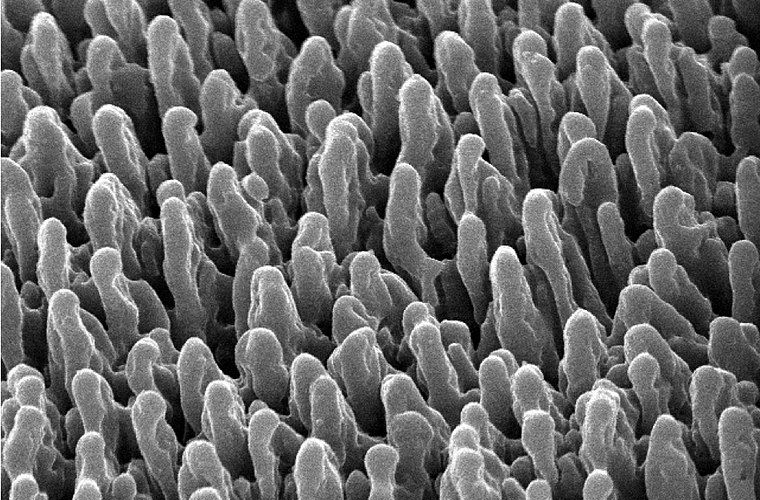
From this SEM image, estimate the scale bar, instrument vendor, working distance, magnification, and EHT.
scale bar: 1000 nm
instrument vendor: JEOL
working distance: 19 mm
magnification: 4 K X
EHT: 15 kV